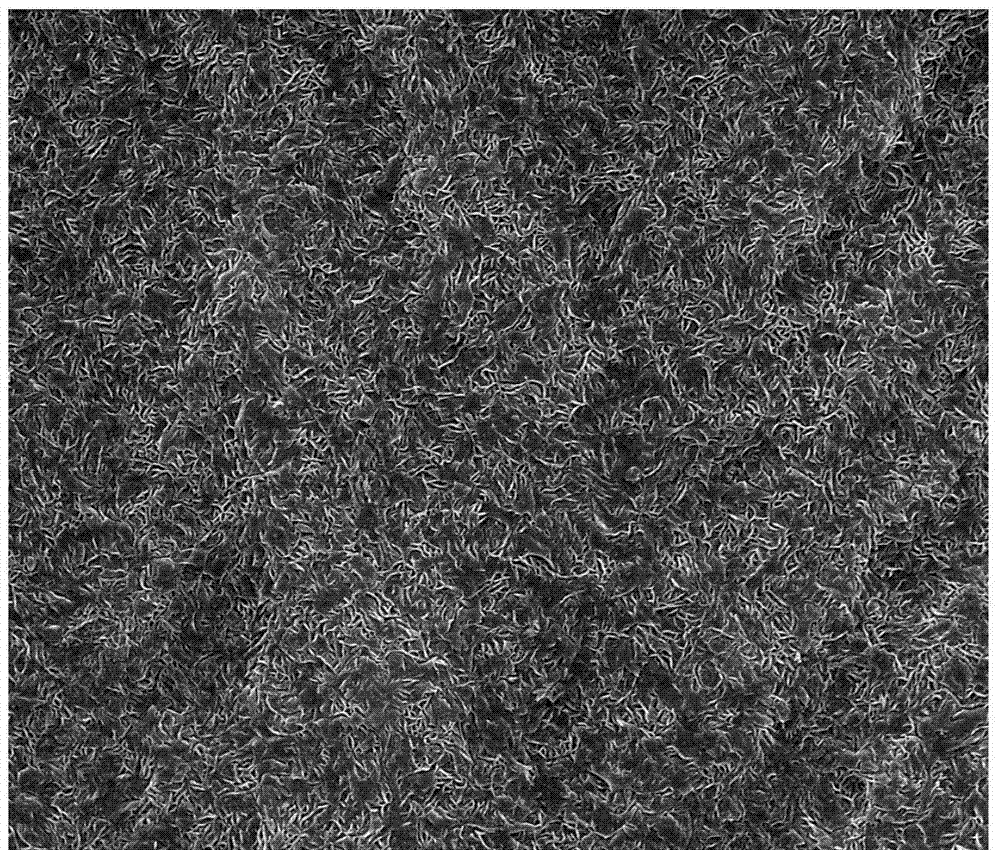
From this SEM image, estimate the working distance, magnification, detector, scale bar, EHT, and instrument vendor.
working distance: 10.8 mm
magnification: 10 K X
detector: SE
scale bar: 10000 nm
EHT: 20 kV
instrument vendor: FEI